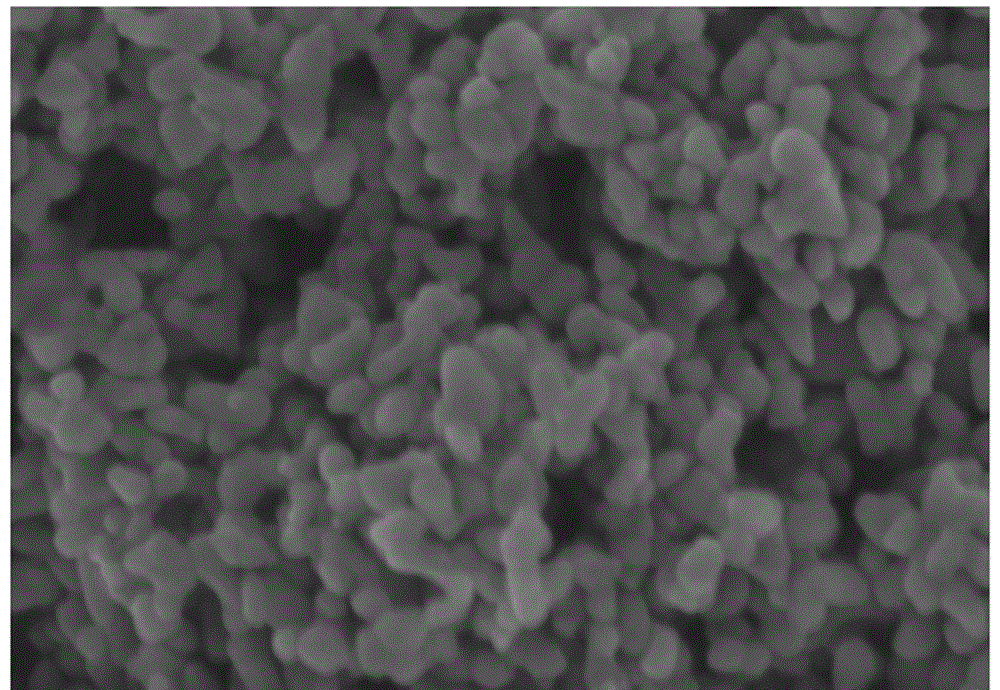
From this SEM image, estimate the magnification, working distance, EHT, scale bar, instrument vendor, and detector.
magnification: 100 K X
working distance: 6 mm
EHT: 3 kV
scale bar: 500 nm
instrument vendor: Hitachi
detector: SE(M)